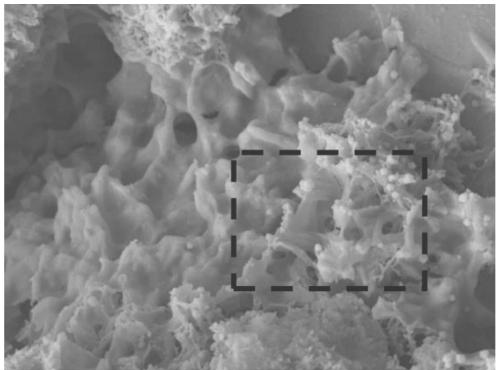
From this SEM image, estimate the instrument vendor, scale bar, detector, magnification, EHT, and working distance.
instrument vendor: JEOL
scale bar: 1000 nm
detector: LEI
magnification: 22 K X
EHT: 10 kV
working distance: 9 mm